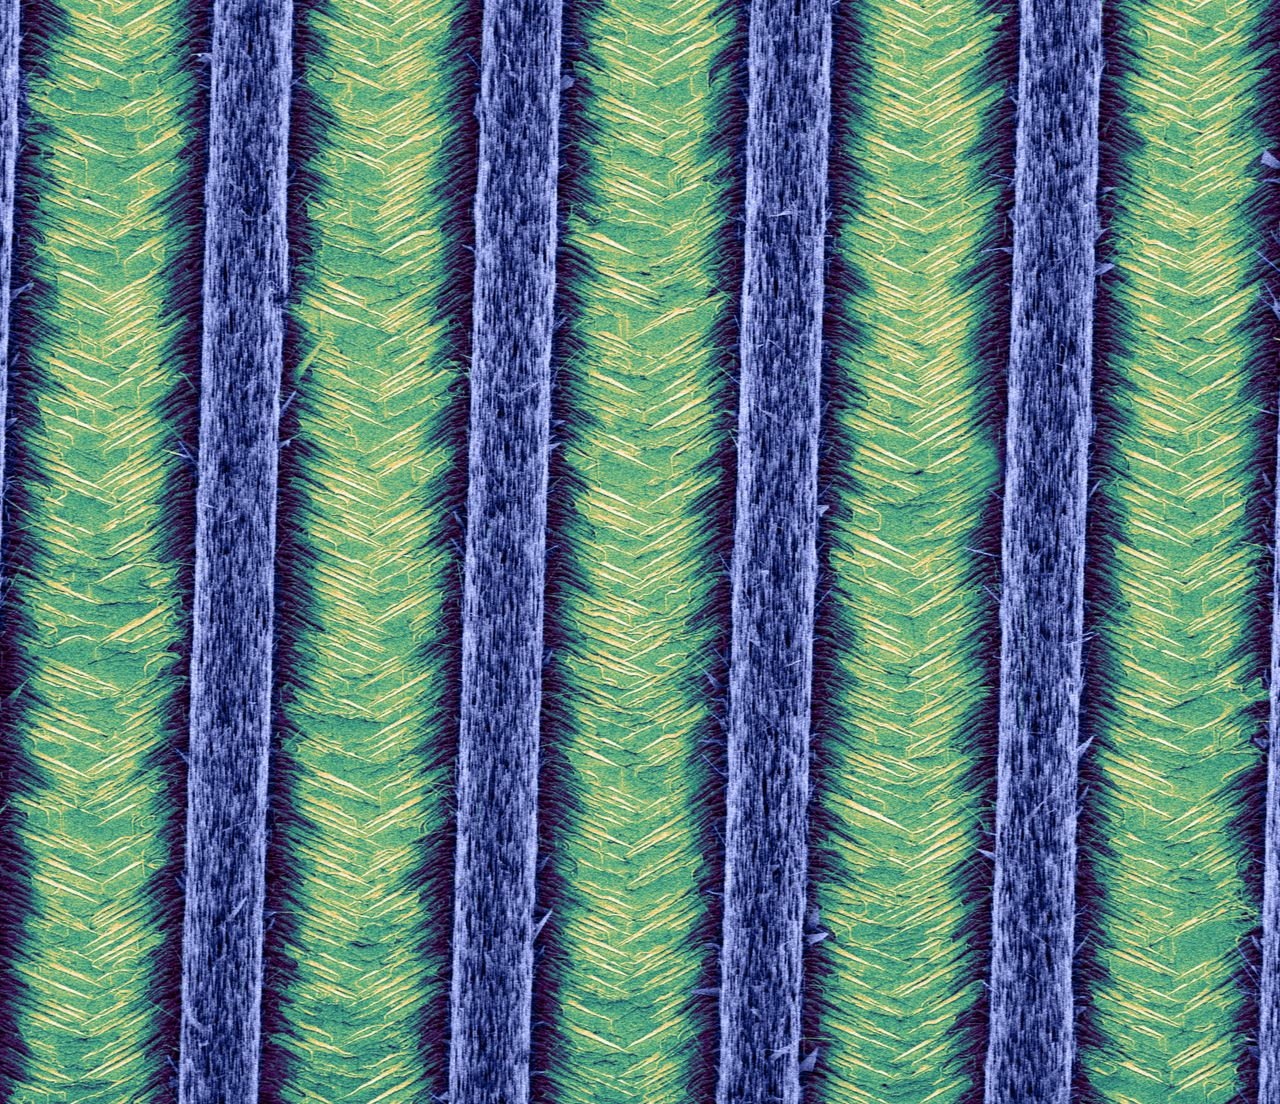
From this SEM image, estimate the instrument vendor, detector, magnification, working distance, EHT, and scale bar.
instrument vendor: FEI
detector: TLD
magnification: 1.25 K X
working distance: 5.2 mm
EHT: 5 kV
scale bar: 50000 nm